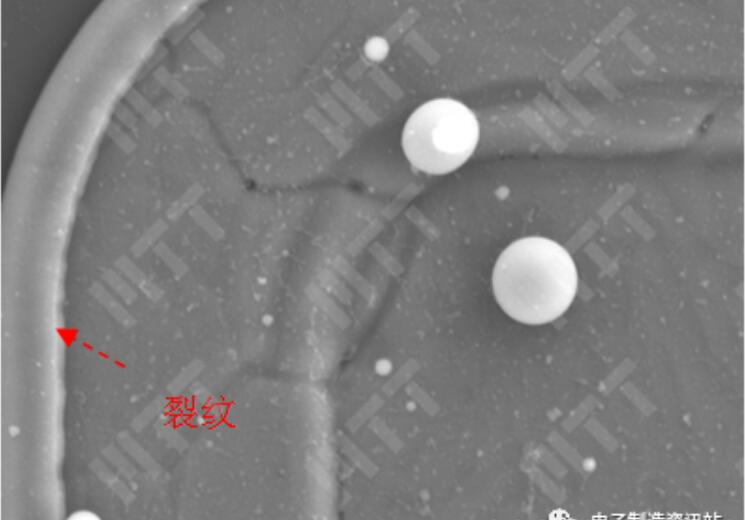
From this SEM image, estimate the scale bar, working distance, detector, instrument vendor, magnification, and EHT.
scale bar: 10000 nm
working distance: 10 mm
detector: SE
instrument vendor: Hitachi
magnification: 4 K X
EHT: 15 kV